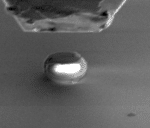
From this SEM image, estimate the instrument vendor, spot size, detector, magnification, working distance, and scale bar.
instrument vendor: FEI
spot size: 3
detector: SE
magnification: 5 K X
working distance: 10 mm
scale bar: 5000 nm